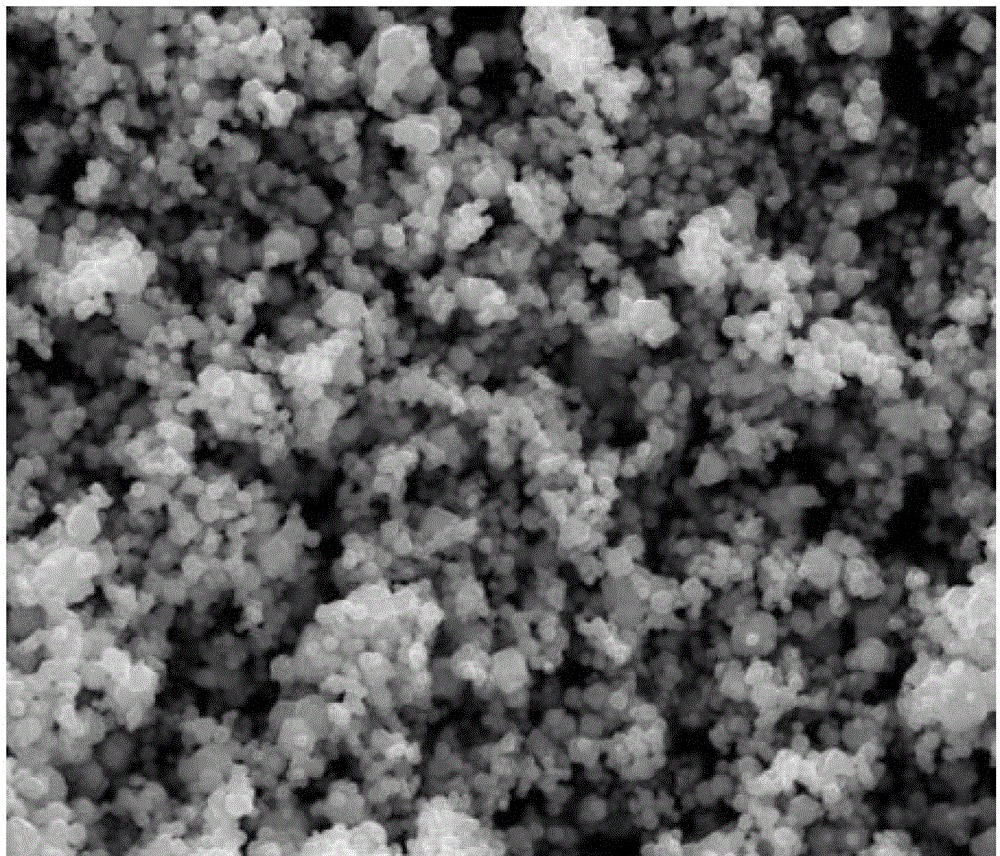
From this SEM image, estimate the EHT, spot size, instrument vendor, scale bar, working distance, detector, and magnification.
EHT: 20 kV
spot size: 2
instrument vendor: FEI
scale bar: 5000 nm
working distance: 10.1 mm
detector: ETD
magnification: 20 K X